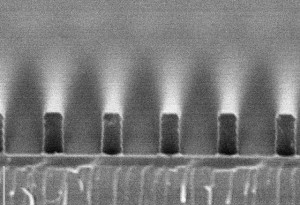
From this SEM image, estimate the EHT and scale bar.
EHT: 10 kV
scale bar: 1000 nm